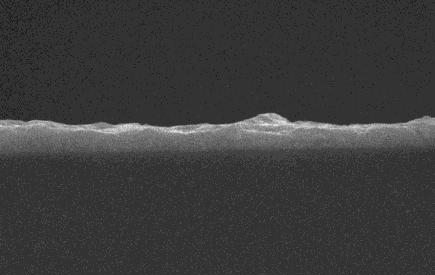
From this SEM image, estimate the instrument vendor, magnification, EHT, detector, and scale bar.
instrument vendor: JEOL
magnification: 5 K X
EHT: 20 kV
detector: SEI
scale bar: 5000 nm